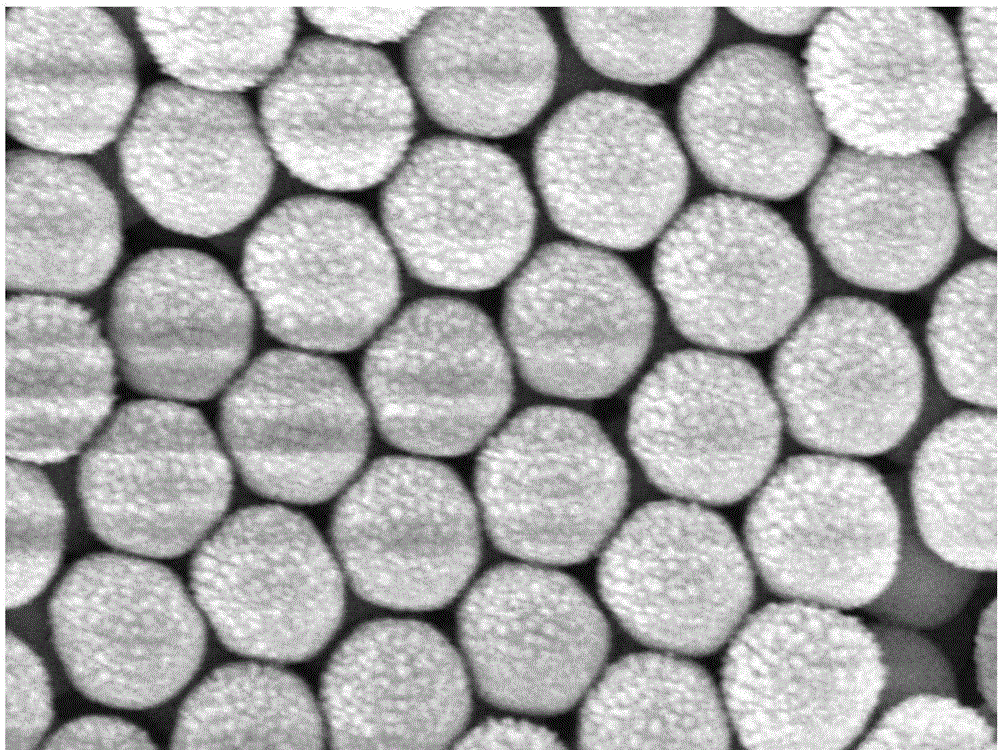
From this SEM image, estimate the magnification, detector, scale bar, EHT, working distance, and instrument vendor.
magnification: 70 K X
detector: SEI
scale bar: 100 nm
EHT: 10 kV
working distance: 5.5 mm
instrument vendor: JEOL